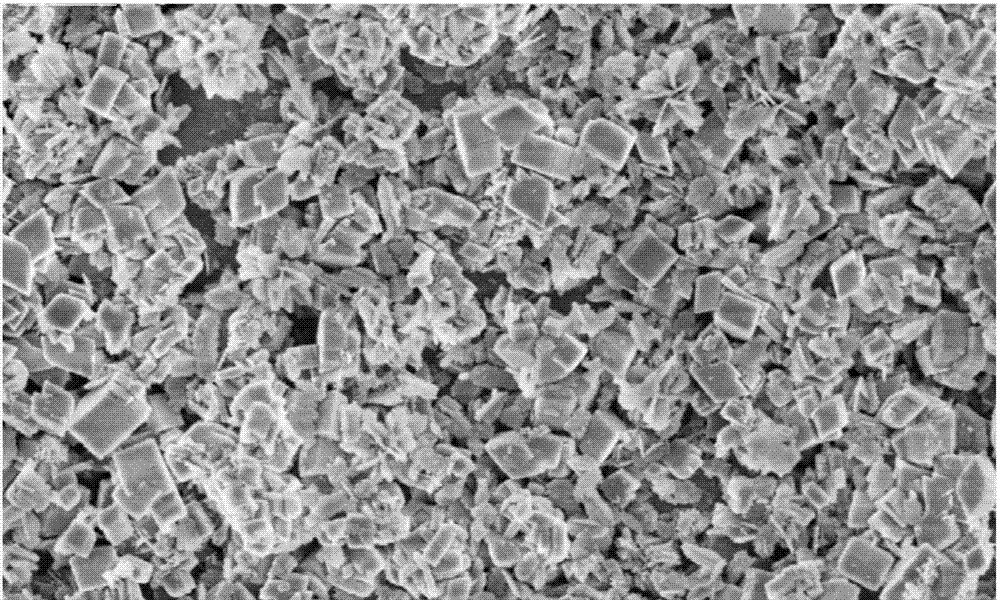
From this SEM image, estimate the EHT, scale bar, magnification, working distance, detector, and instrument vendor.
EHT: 2 kV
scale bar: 2000 nm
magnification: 20 K X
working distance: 8.8 mm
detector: SE(U)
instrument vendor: Hitachi